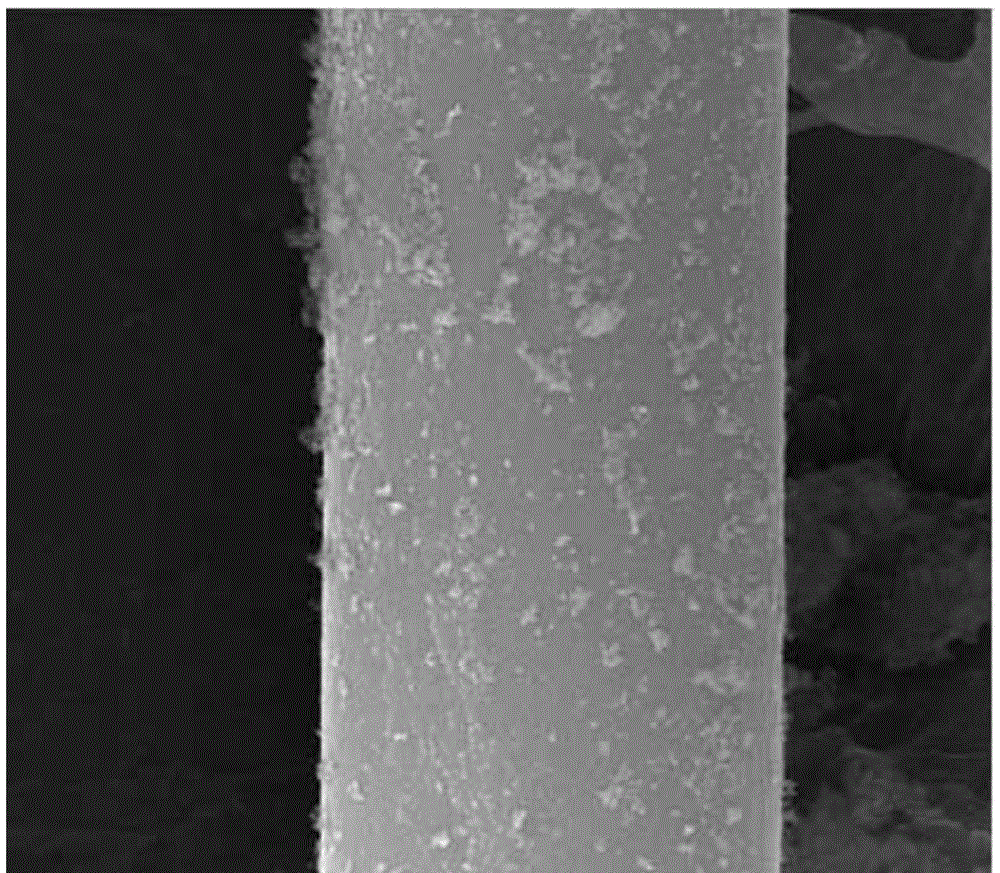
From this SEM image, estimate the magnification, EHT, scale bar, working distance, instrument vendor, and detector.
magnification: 20 K X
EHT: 30 kV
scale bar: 5000 nm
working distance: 10.8 mm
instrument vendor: FEI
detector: SE